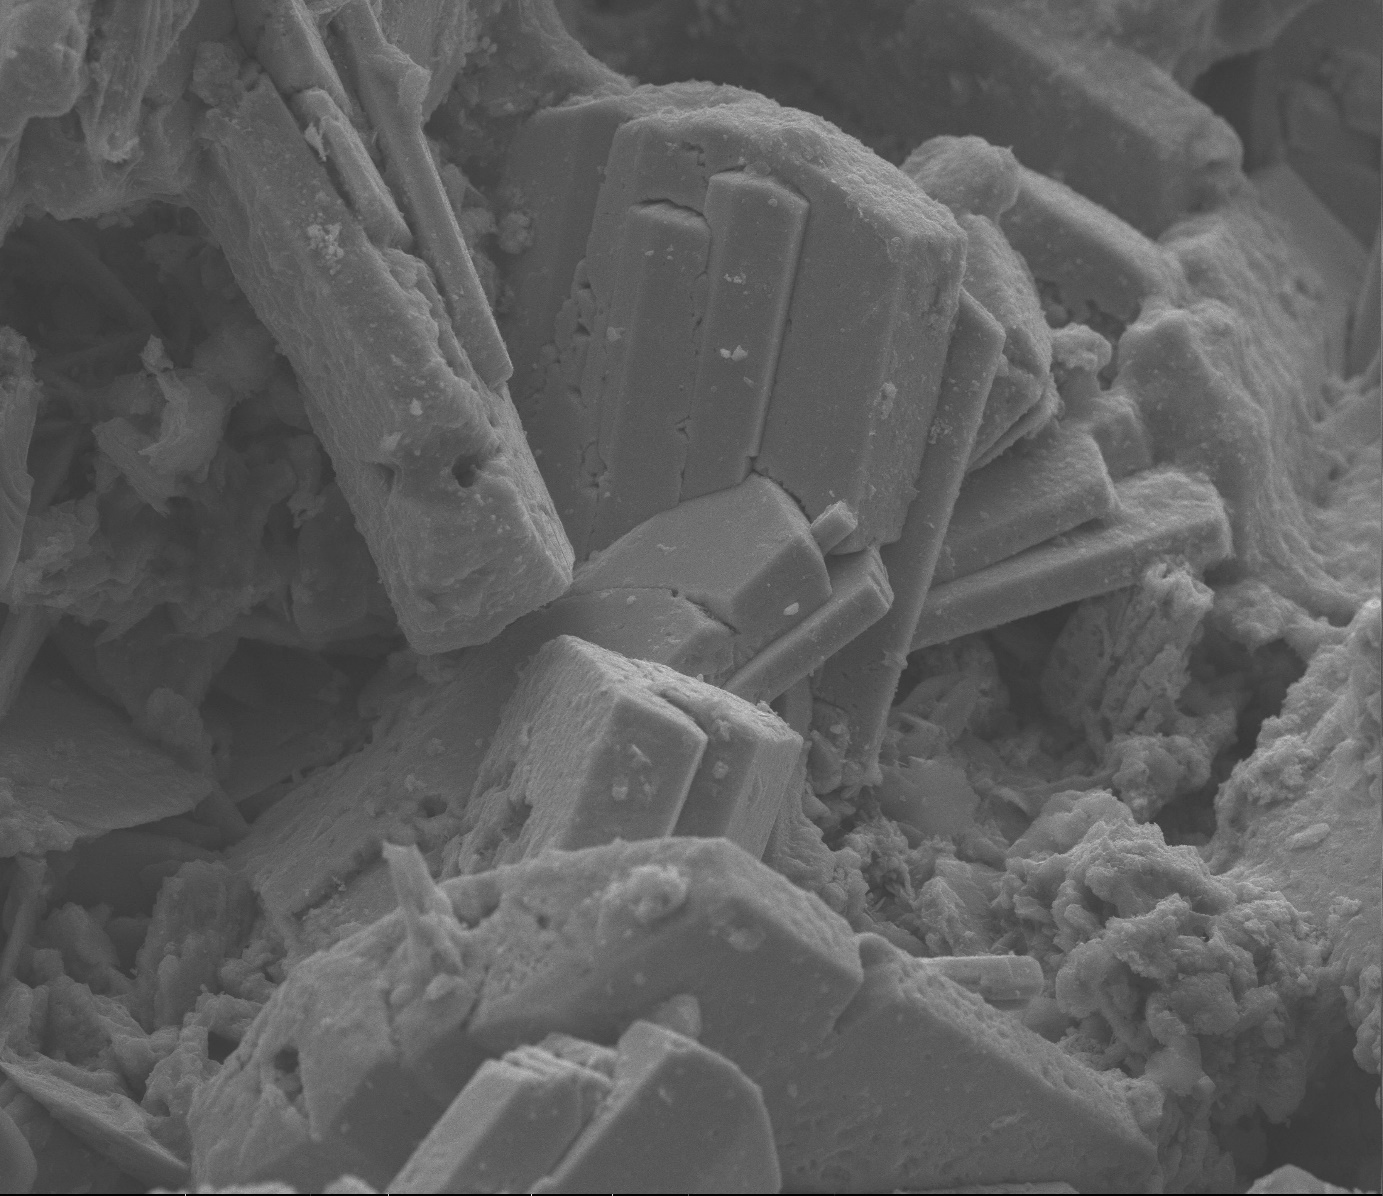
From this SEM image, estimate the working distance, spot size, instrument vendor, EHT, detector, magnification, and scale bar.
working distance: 12.3 mm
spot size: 3.5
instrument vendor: FEI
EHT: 30 kV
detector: ETD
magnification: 0.875 K X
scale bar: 100000 nm